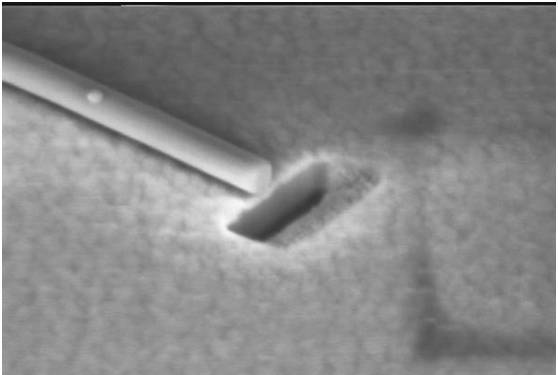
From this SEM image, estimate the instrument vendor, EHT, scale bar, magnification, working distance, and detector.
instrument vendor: Zeiss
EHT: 2 kV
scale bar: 100 nm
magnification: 188.39 K X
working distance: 5 mm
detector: InLens